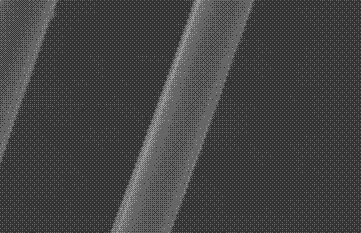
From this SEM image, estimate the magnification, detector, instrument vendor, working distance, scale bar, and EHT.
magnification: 3 K X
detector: SE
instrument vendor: FEI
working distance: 9.8 mm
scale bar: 10000 nm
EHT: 20 kV